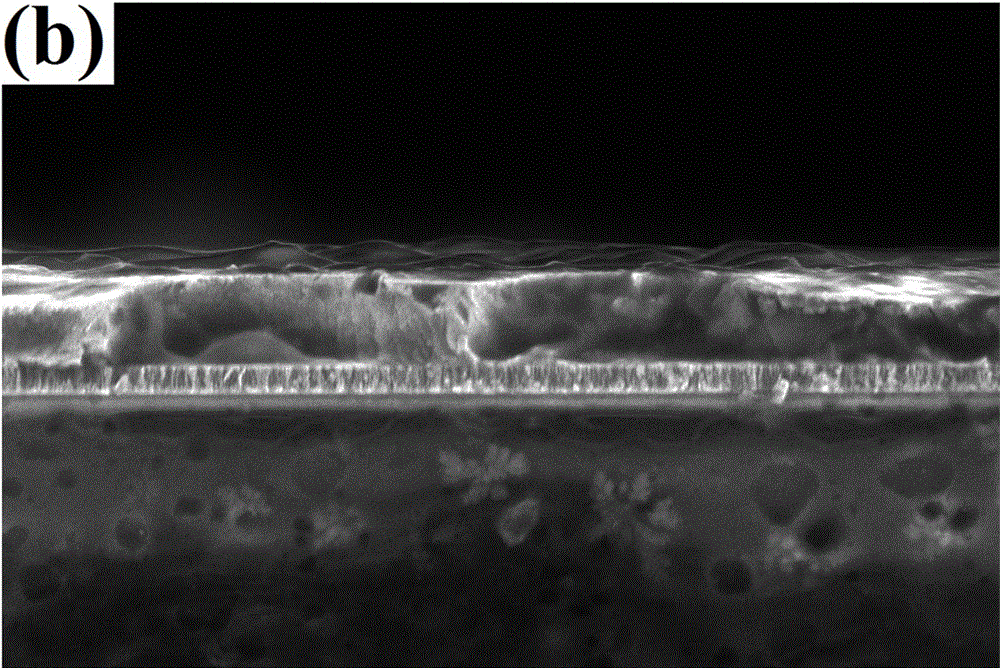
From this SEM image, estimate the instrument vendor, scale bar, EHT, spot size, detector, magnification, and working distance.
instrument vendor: FEI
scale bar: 5000 nm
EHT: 5 kV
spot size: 3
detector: TLD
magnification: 25 K X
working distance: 5 mm